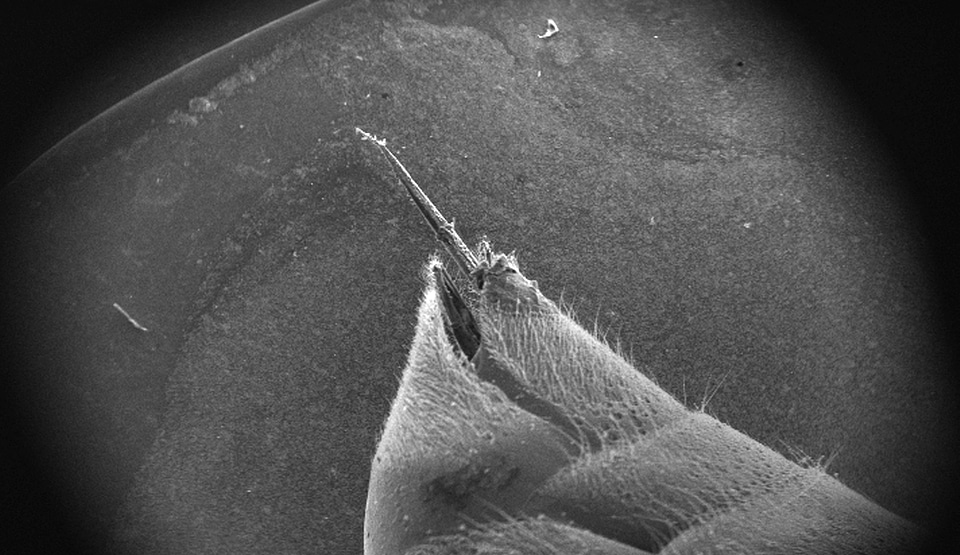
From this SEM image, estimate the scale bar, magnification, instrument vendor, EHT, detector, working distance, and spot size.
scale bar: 1000000 nm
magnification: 0.023 K X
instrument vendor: FEI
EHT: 30 kV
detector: SE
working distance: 7.4 mm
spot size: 3